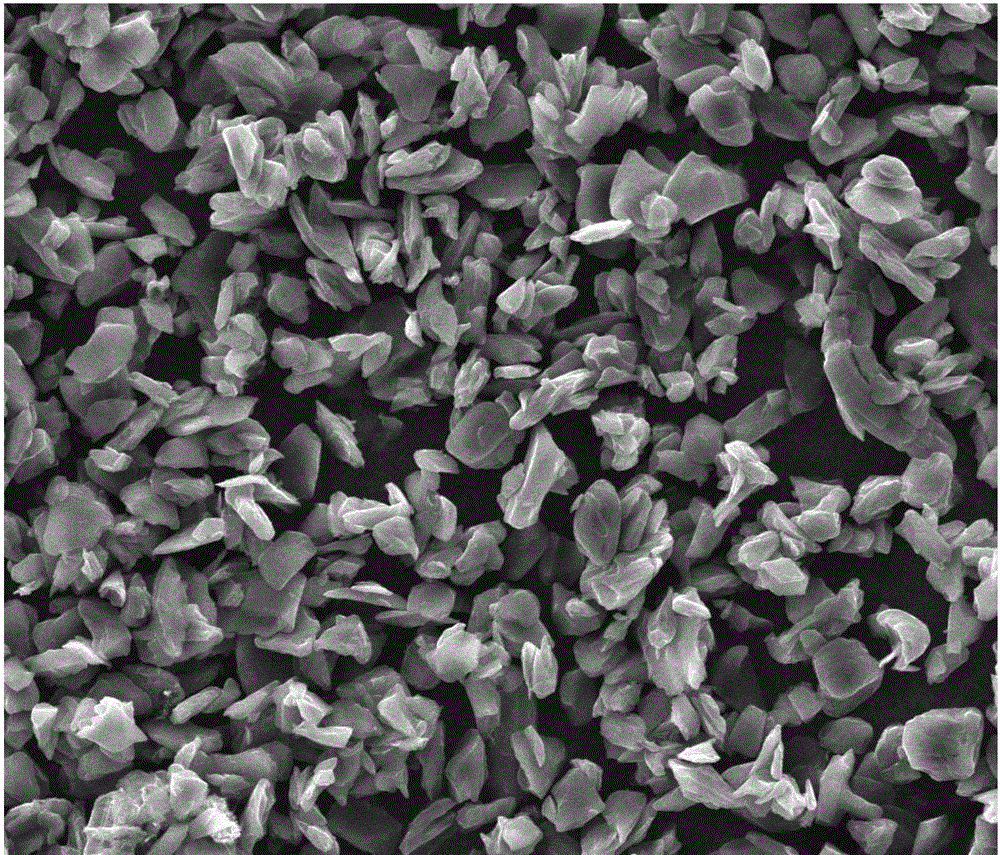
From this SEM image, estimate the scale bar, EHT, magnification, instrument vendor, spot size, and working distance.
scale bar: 50000 nm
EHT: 20 kV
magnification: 1 K X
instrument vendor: FEI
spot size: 5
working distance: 9.9 mm